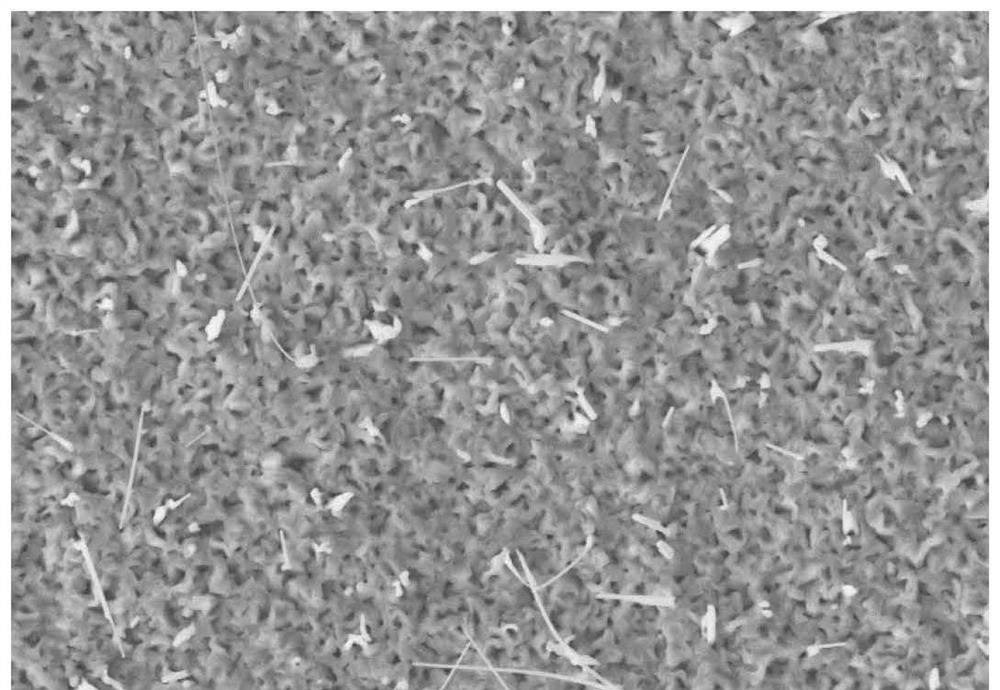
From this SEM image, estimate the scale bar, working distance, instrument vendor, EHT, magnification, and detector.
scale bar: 2000 nm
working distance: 10.1 mm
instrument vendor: Zeiss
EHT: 15 kV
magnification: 3 K X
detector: SE2